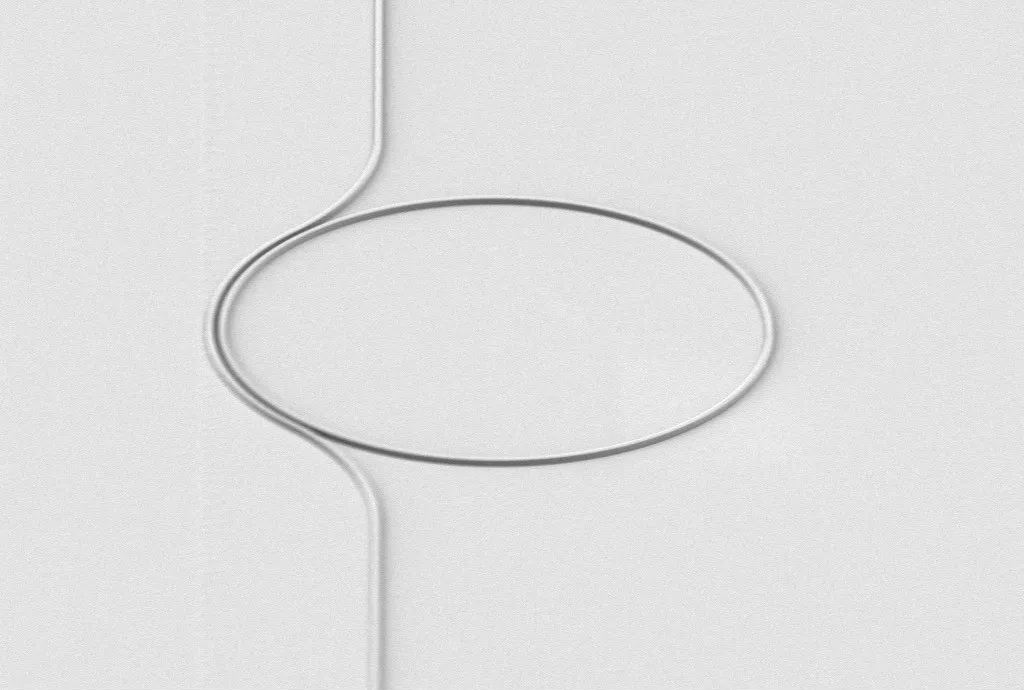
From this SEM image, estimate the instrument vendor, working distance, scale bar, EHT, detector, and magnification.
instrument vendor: Zeiss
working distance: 20.1 mm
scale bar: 10000 nm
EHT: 3 kV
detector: SE2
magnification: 3 K X